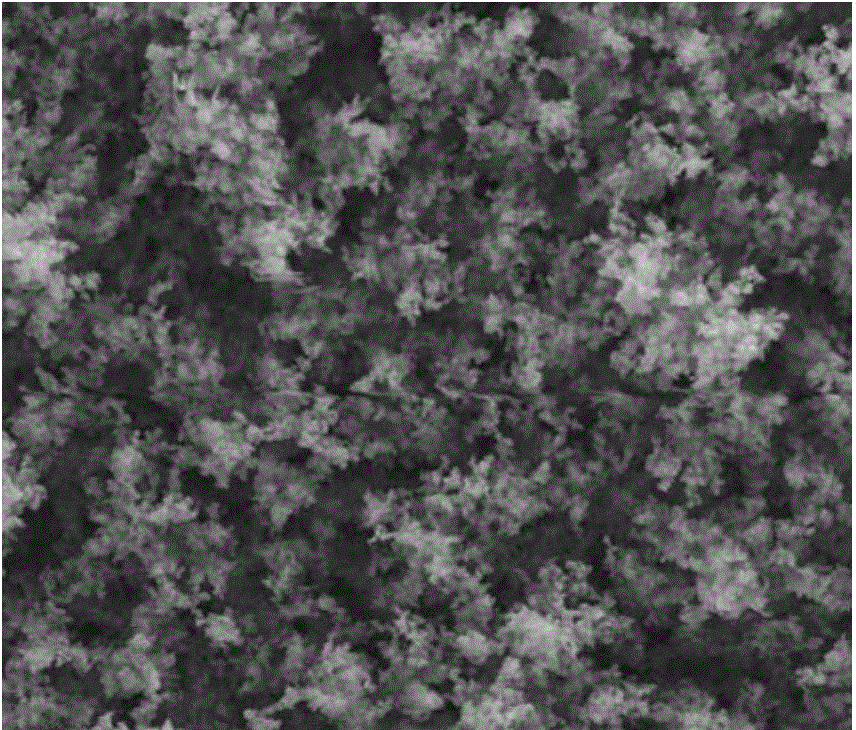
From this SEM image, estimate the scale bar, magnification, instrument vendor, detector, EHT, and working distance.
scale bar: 5000 nm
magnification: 20 K X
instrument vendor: FEI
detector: SE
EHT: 20 kV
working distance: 11.7 mm